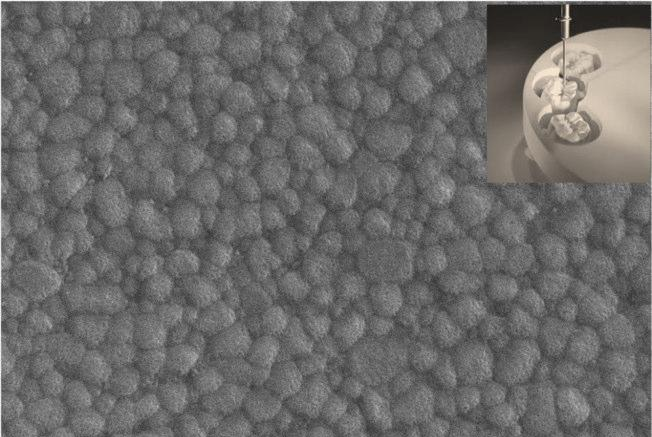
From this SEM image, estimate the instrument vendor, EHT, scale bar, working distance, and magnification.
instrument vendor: Zeiss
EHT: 25 kV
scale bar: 1000 nm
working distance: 10 mm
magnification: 34.34 K X